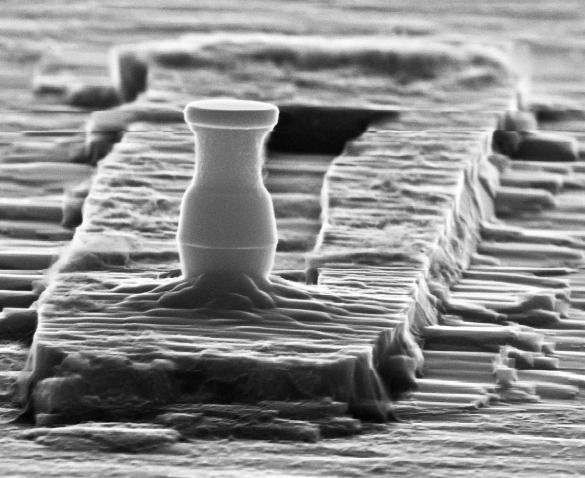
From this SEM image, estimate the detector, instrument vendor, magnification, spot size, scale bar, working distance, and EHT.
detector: SE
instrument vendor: FEI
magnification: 28.077 K X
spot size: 4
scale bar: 1000 nm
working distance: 12.6 mm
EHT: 30 kV